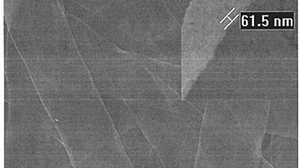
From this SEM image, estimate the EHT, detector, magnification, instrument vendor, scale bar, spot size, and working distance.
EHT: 20 kV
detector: SE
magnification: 5 K X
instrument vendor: FEI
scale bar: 5000 nm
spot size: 3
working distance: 9.9 mm